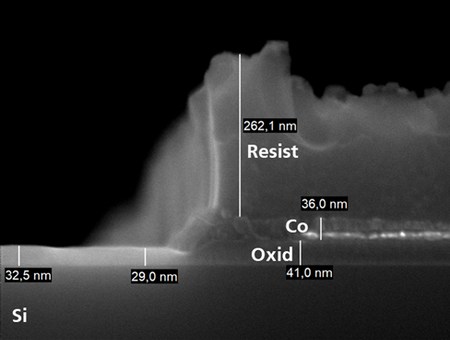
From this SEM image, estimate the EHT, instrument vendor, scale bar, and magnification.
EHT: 10 kV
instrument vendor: Zeiss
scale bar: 100 nm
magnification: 150 K X